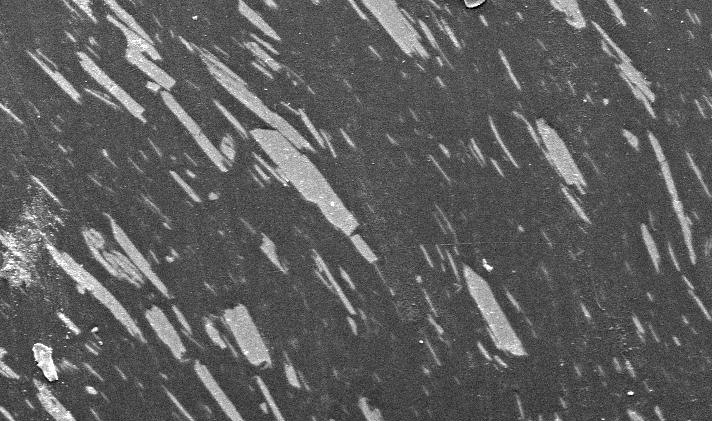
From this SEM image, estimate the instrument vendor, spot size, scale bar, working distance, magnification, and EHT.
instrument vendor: FEI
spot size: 5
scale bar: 50000 nm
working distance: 5.9 mm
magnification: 0.5 K X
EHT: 20 kV